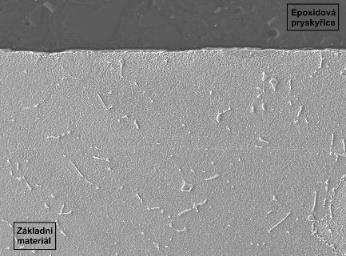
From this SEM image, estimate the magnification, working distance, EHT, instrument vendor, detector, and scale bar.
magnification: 0.5 K X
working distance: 8 mm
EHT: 15 kV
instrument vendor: JEOL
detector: LEI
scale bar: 10000 nm